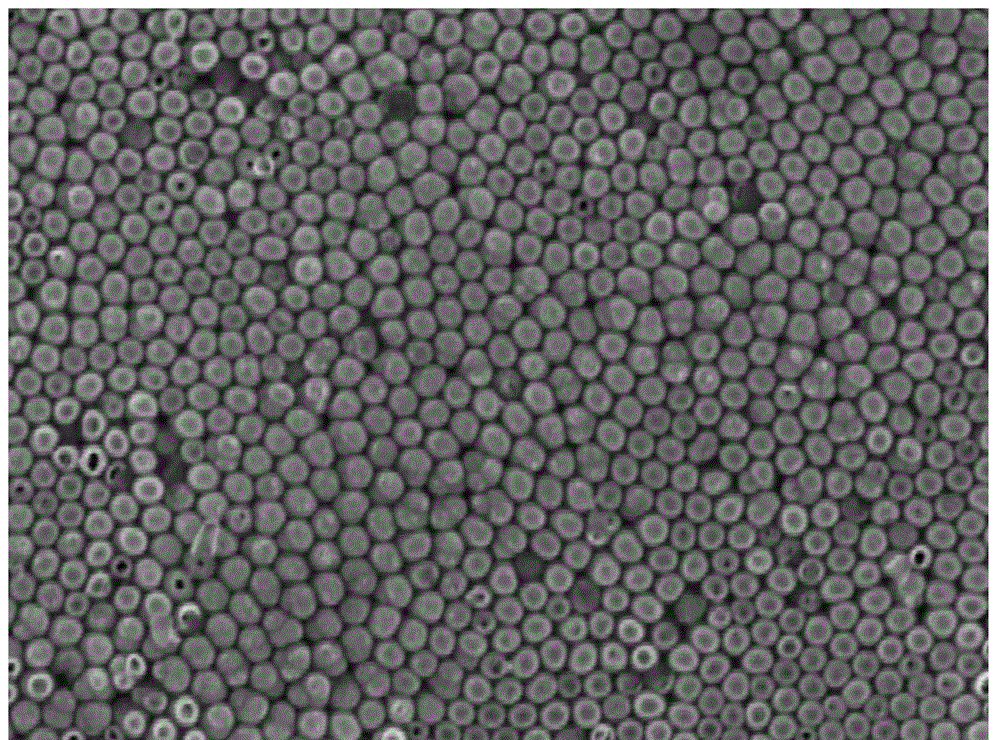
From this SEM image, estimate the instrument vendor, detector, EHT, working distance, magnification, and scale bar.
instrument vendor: Tescan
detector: SE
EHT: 15 kV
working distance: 14.92 mm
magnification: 10 K X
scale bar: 2000 nm